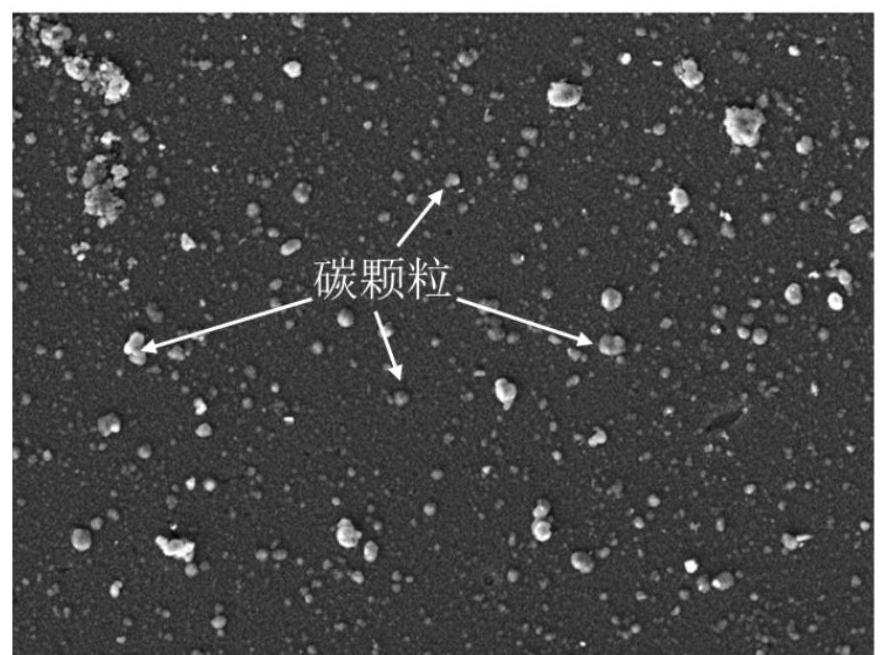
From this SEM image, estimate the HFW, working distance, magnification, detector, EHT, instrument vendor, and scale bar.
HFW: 55.4 µm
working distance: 20.45 mm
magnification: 5 K X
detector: SE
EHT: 7 kV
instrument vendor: Tescan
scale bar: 10000 nm